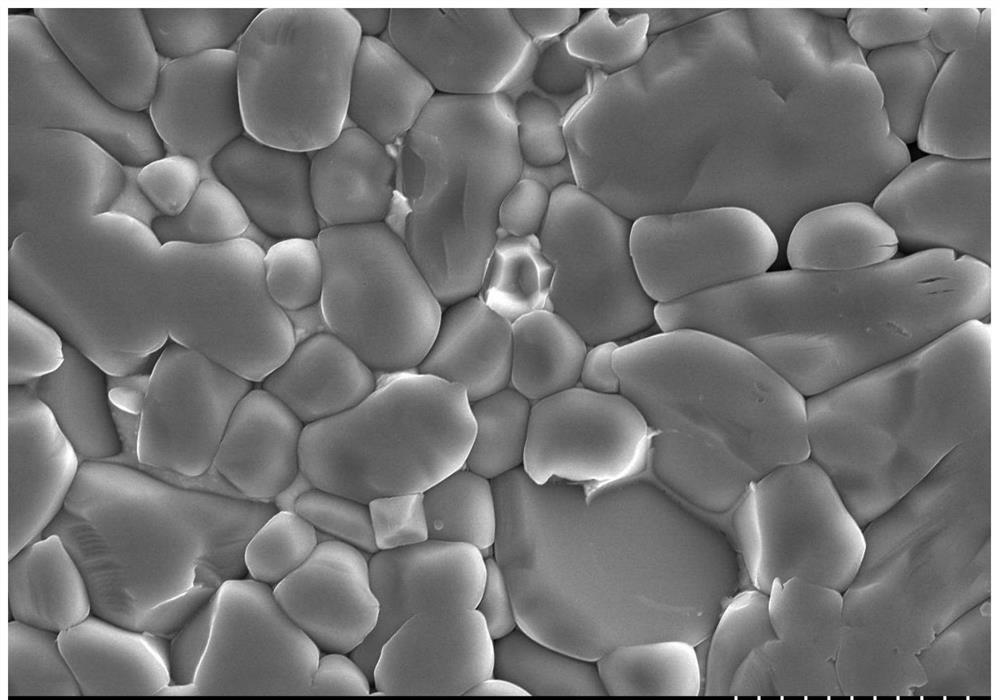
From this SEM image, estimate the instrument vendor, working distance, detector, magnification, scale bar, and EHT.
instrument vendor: Hitachi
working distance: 15.3 mm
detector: SE(UL)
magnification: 3 K X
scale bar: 10000 nm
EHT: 20 kV